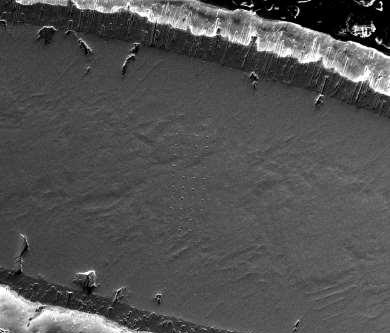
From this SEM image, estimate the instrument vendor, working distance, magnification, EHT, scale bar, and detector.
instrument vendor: FEI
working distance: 9.9 mm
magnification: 0.2 K X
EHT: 20 kV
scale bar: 300000 nm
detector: ETD